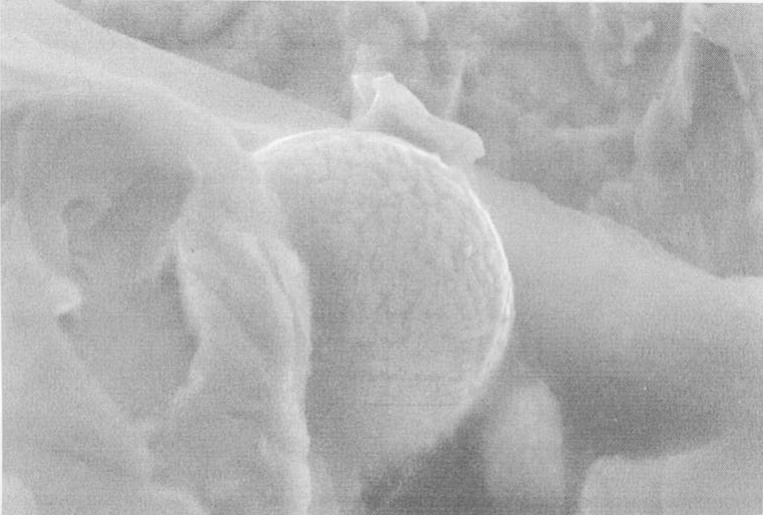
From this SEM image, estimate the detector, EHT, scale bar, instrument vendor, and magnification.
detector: SEI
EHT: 30 kV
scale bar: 10000 nm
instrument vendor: JEOL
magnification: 2.5 K X